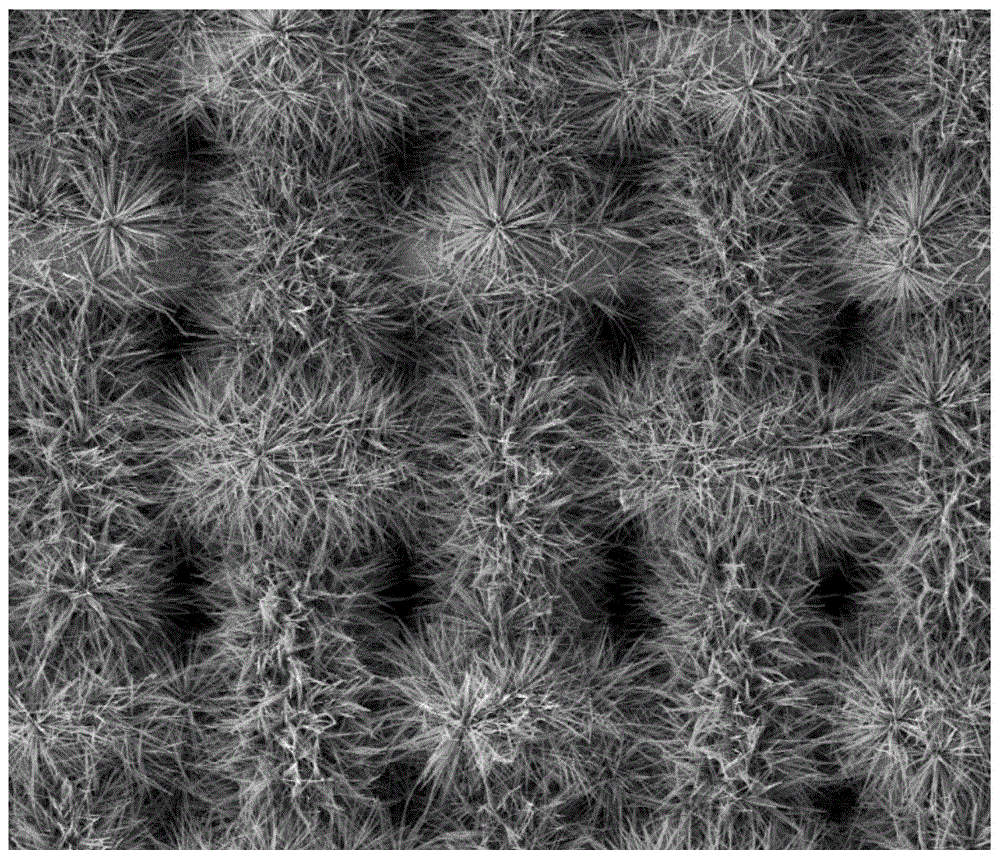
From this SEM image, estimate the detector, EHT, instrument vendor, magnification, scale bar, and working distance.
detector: ETD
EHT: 10 kV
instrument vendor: FEI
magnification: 1 K X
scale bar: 100000 nm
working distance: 10.5 mm